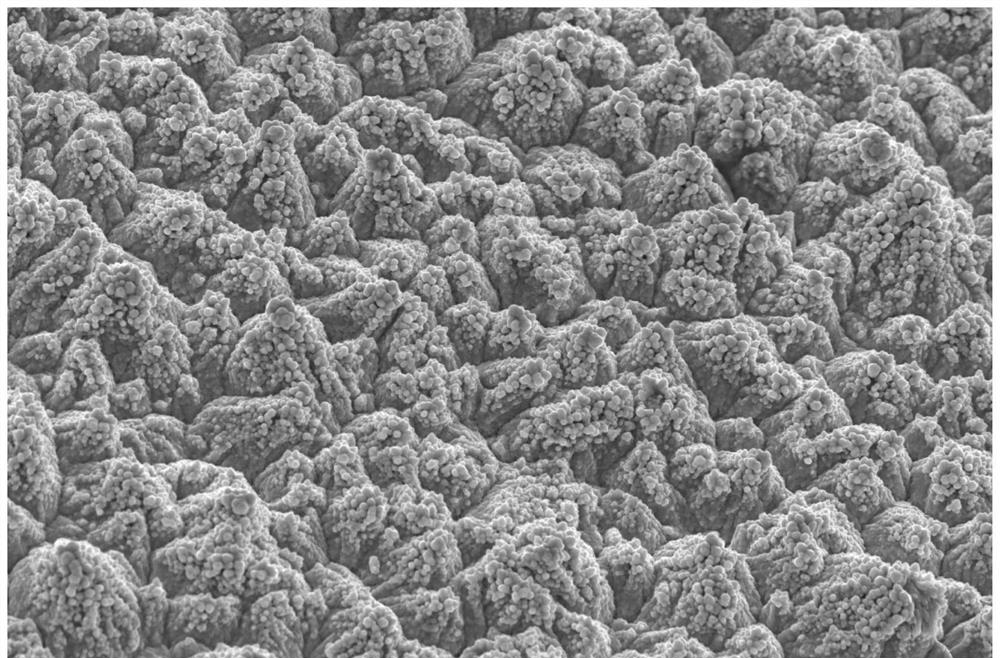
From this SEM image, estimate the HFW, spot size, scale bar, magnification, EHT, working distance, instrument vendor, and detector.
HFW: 82.9 µm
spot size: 3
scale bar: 30000 nm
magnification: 5 K X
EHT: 20 kV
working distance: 10.3 mm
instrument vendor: FEI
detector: ETD